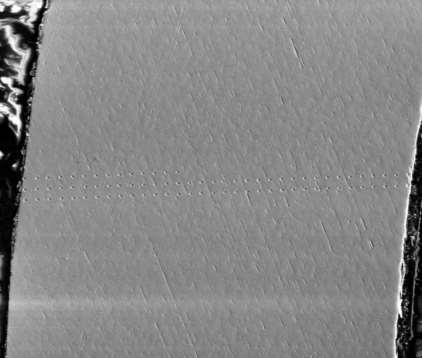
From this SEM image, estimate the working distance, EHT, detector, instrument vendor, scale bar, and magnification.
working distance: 10 mm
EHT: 20 kV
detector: ETD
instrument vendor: FEI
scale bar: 300000 nm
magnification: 0.2 K X